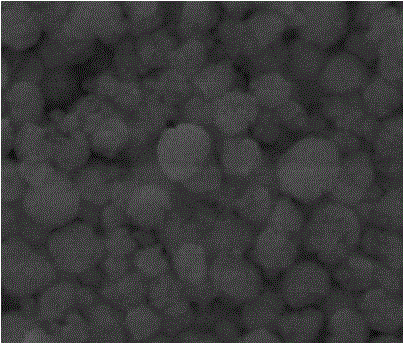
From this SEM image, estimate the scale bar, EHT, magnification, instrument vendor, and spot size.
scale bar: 1000 nm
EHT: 20 kV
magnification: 100 K X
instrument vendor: FEI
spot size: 2.5